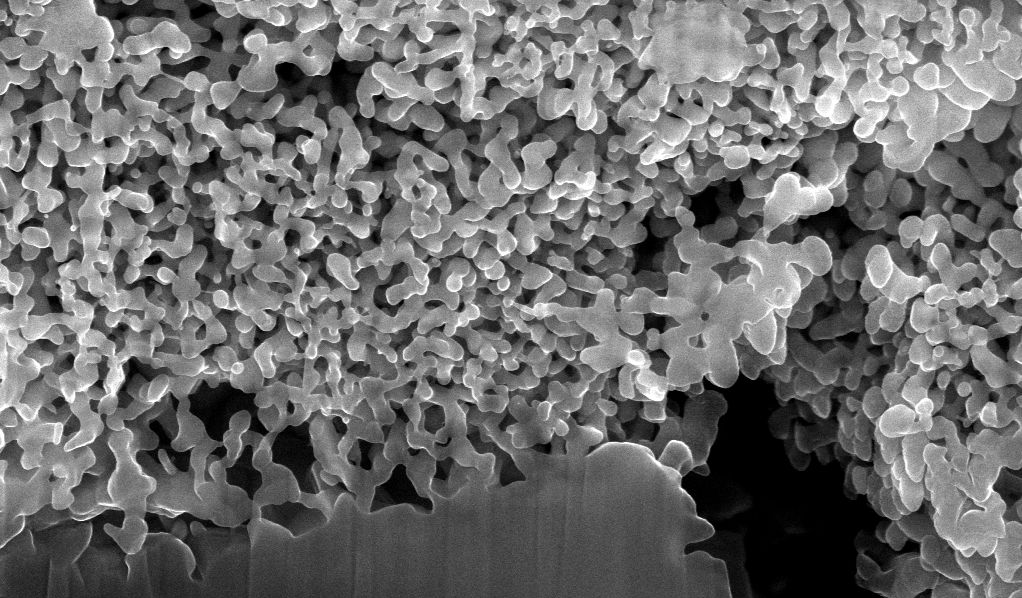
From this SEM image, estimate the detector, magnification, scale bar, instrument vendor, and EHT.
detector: TLD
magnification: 40.026 K X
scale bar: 1000 nm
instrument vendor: FEI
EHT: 15 kV